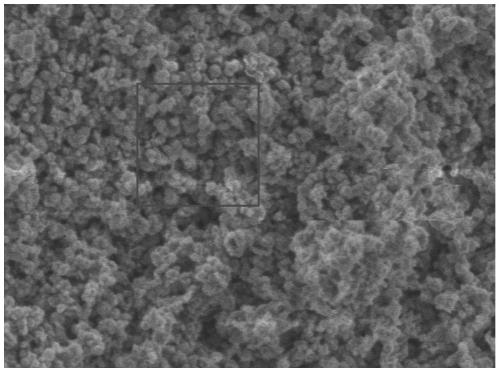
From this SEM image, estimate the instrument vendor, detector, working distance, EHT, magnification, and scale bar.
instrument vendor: JEOL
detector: LED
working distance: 8 mm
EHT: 1 kV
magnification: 30 K X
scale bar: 100 nm